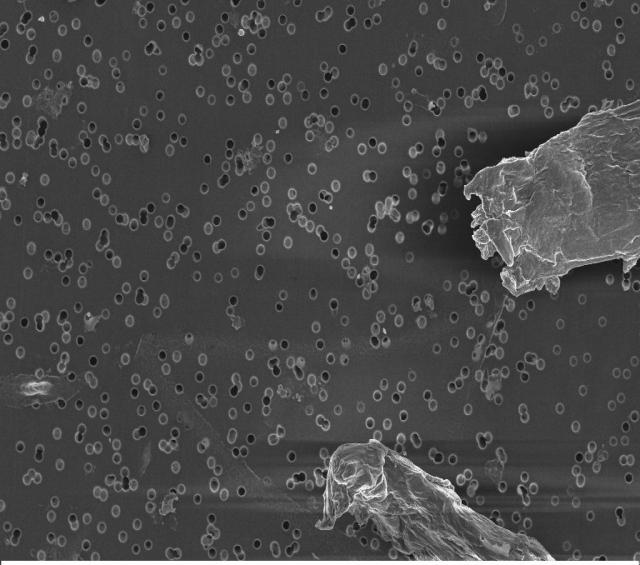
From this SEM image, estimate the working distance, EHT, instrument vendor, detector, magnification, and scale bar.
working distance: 2.9 mm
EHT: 3 kV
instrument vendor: Zeiss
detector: InLens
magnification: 5 K X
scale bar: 10000 nm